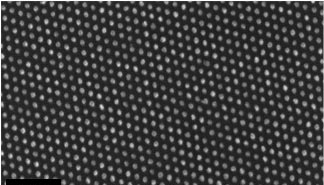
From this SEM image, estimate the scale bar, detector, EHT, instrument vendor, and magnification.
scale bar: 200 nm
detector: InLens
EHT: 5 kV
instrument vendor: Zeiss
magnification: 100 K X